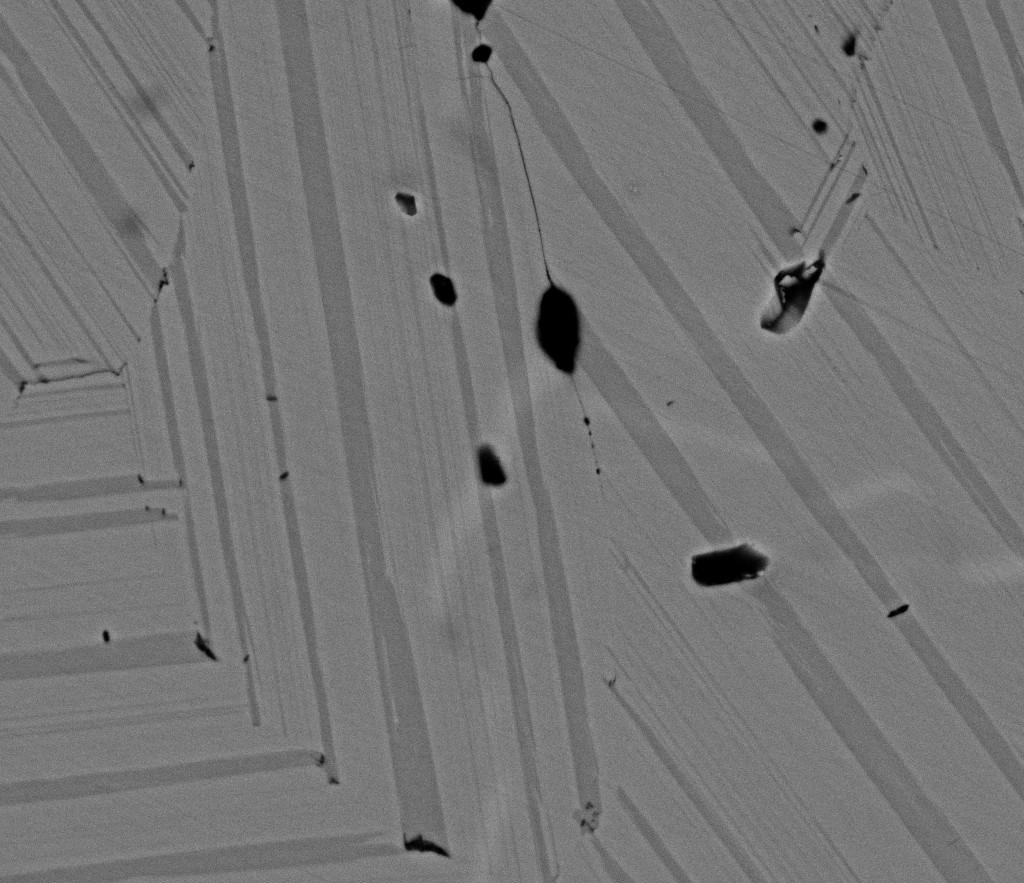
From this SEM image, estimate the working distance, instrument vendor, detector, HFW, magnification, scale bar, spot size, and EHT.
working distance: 6.6 mm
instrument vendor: FEI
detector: BSED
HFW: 74.6 µm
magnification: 4 K X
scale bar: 20000 nm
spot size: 4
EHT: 18 kV